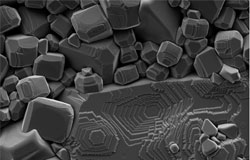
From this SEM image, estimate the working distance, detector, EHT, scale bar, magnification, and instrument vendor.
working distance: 8 mm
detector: SE2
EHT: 5 kV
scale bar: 2000 nm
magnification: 12.5 K X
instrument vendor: Zeiss